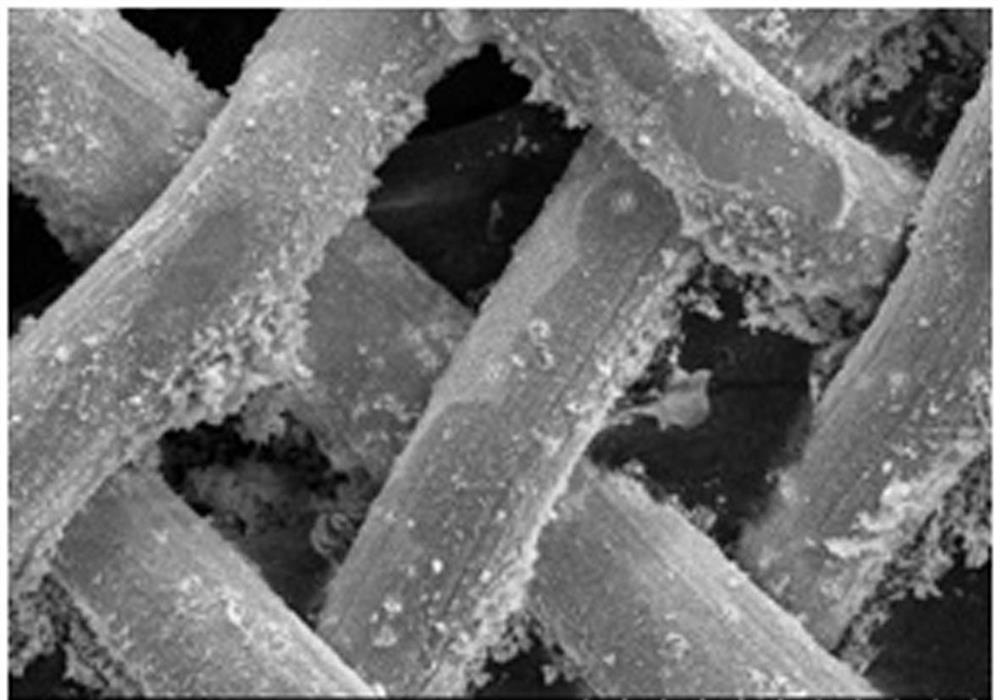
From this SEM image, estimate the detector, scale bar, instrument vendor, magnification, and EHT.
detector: SE(M,LA0)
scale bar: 100000 nm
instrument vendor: Hitachi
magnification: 0.5 K X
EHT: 10 kV